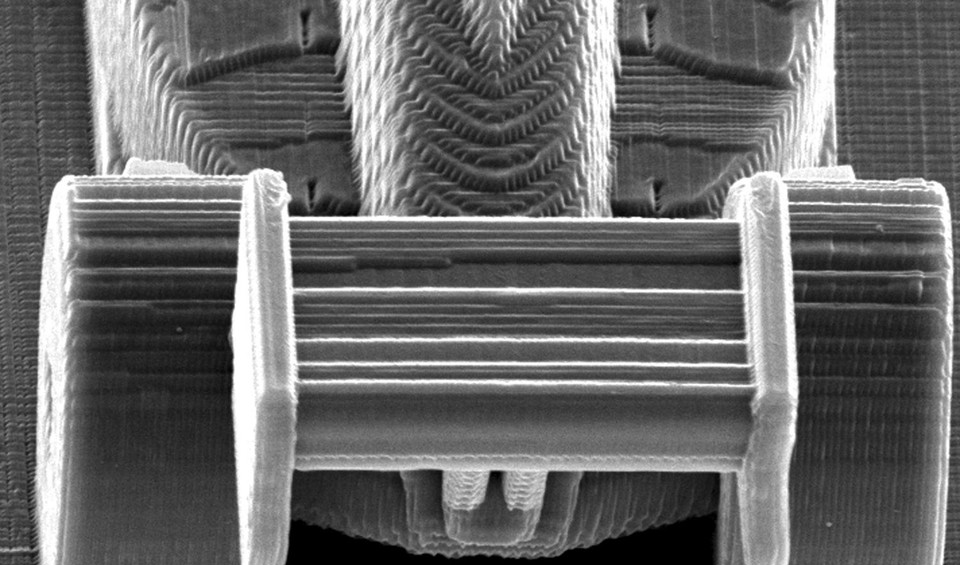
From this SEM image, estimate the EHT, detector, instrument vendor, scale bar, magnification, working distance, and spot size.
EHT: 20 kV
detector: SE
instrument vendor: FEI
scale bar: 20000 nm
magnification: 0.922 K X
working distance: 27.2 mm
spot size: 4